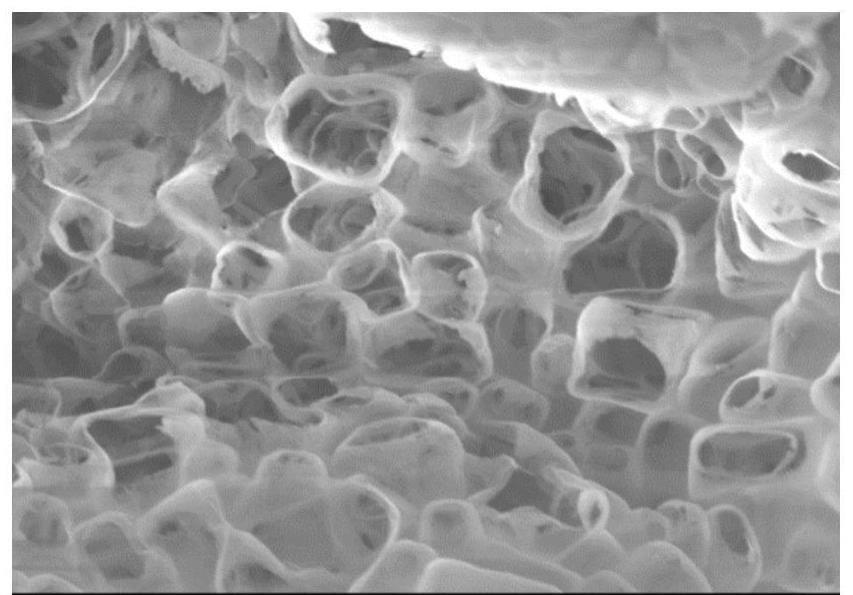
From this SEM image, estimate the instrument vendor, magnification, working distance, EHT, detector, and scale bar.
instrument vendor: Zeiss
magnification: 20 K X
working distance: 6 mm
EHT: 2 kV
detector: InLens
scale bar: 500 nm